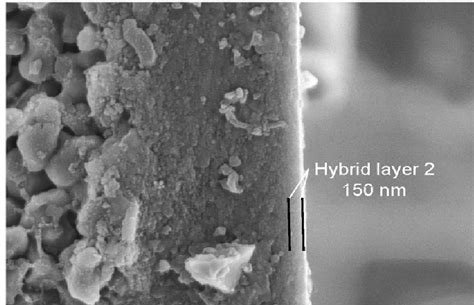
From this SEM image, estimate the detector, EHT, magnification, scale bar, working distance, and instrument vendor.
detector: SEI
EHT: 5 kV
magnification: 30 K X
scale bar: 100 nm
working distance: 13 mm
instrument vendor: JEOL